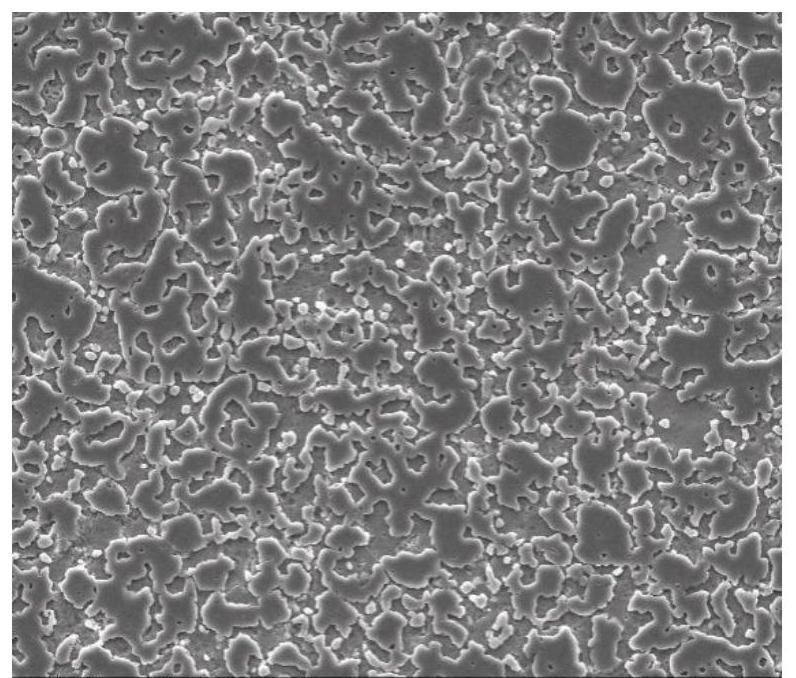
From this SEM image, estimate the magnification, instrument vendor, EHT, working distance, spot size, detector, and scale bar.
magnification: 1 K X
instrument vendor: FEI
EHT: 20 kV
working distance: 13.1 mm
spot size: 4.5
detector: ETD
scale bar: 100000 nm